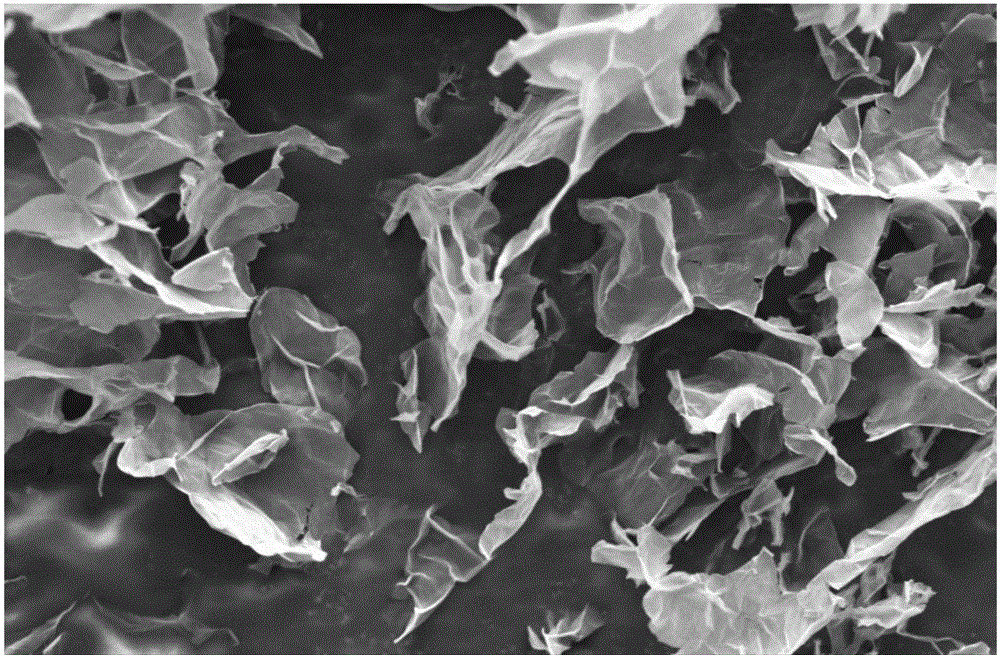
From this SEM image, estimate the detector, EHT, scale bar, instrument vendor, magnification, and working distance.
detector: SE1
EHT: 5 kV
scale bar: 2000 nm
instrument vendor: Zeiss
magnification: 2.5 K X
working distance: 8 mm